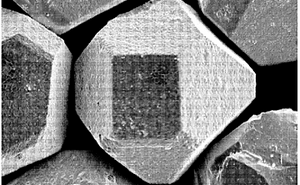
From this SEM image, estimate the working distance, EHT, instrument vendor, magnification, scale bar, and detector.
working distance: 10 mm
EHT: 10 kV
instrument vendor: JEOL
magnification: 0.55 K X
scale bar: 20000 nm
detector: SEI